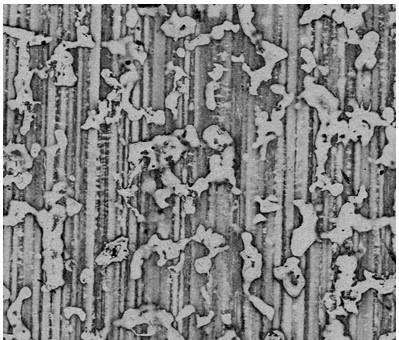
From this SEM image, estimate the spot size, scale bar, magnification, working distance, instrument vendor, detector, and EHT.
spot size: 2.5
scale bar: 200000 nm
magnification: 0.25 K X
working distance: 9.8 mm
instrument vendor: FEI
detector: vCD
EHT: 15 kV